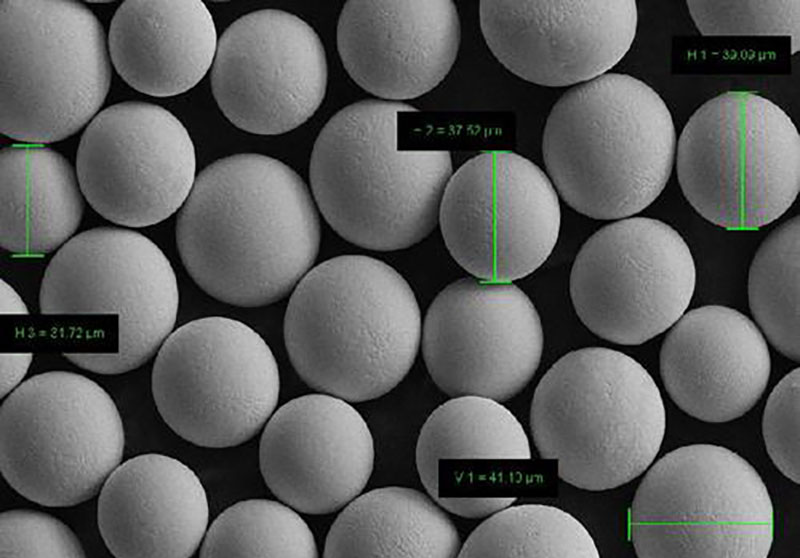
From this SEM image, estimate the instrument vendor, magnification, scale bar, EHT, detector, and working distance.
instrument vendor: Zeiss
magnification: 0.5 K X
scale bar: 10000 nm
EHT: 15 kV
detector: SE2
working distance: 7.6 mm